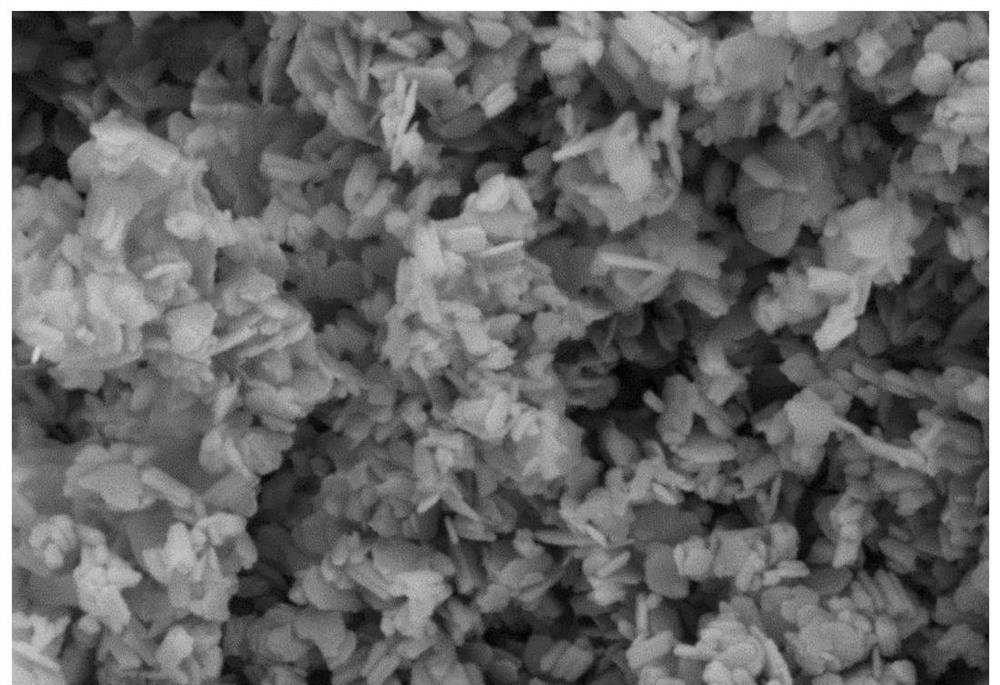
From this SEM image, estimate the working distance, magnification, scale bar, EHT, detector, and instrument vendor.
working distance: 9.2 mm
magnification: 40.5 K X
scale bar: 200 nm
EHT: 5 kV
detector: SE2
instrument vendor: Zeiss